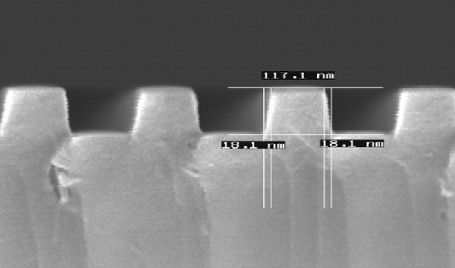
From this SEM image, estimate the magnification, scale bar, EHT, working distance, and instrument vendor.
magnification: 100 K X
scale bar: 200 nm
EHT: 5 kV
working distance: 4 mm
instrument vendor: other